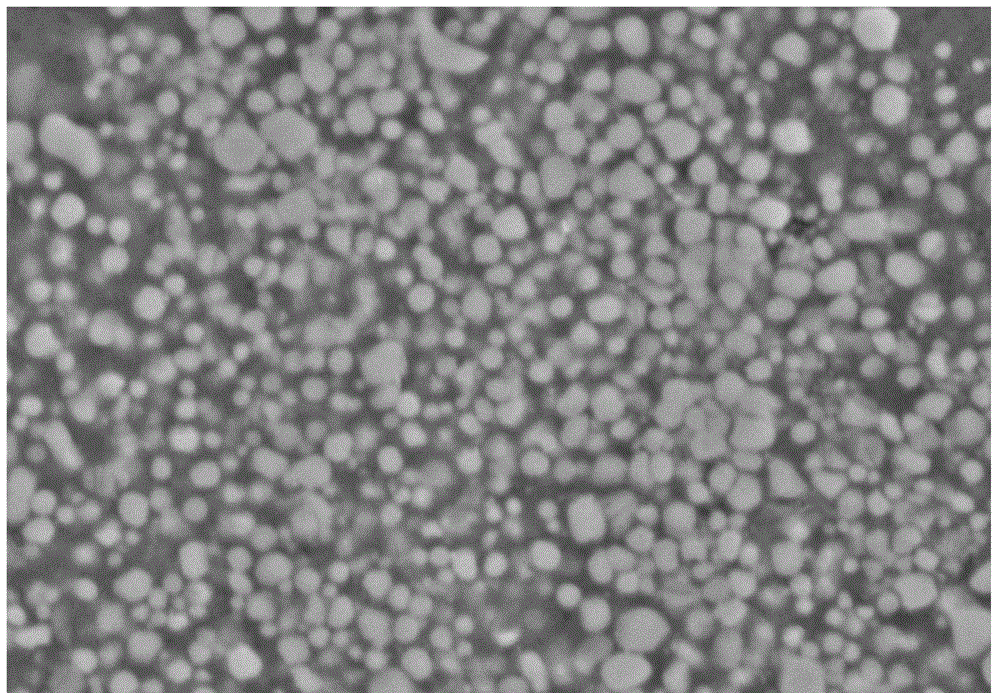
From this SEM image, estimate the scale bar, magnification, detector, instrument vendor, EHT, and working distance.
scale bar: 10000 nm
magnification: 5 K X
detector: SE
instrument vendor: Hitachi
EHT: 15 kV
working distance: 10 mm